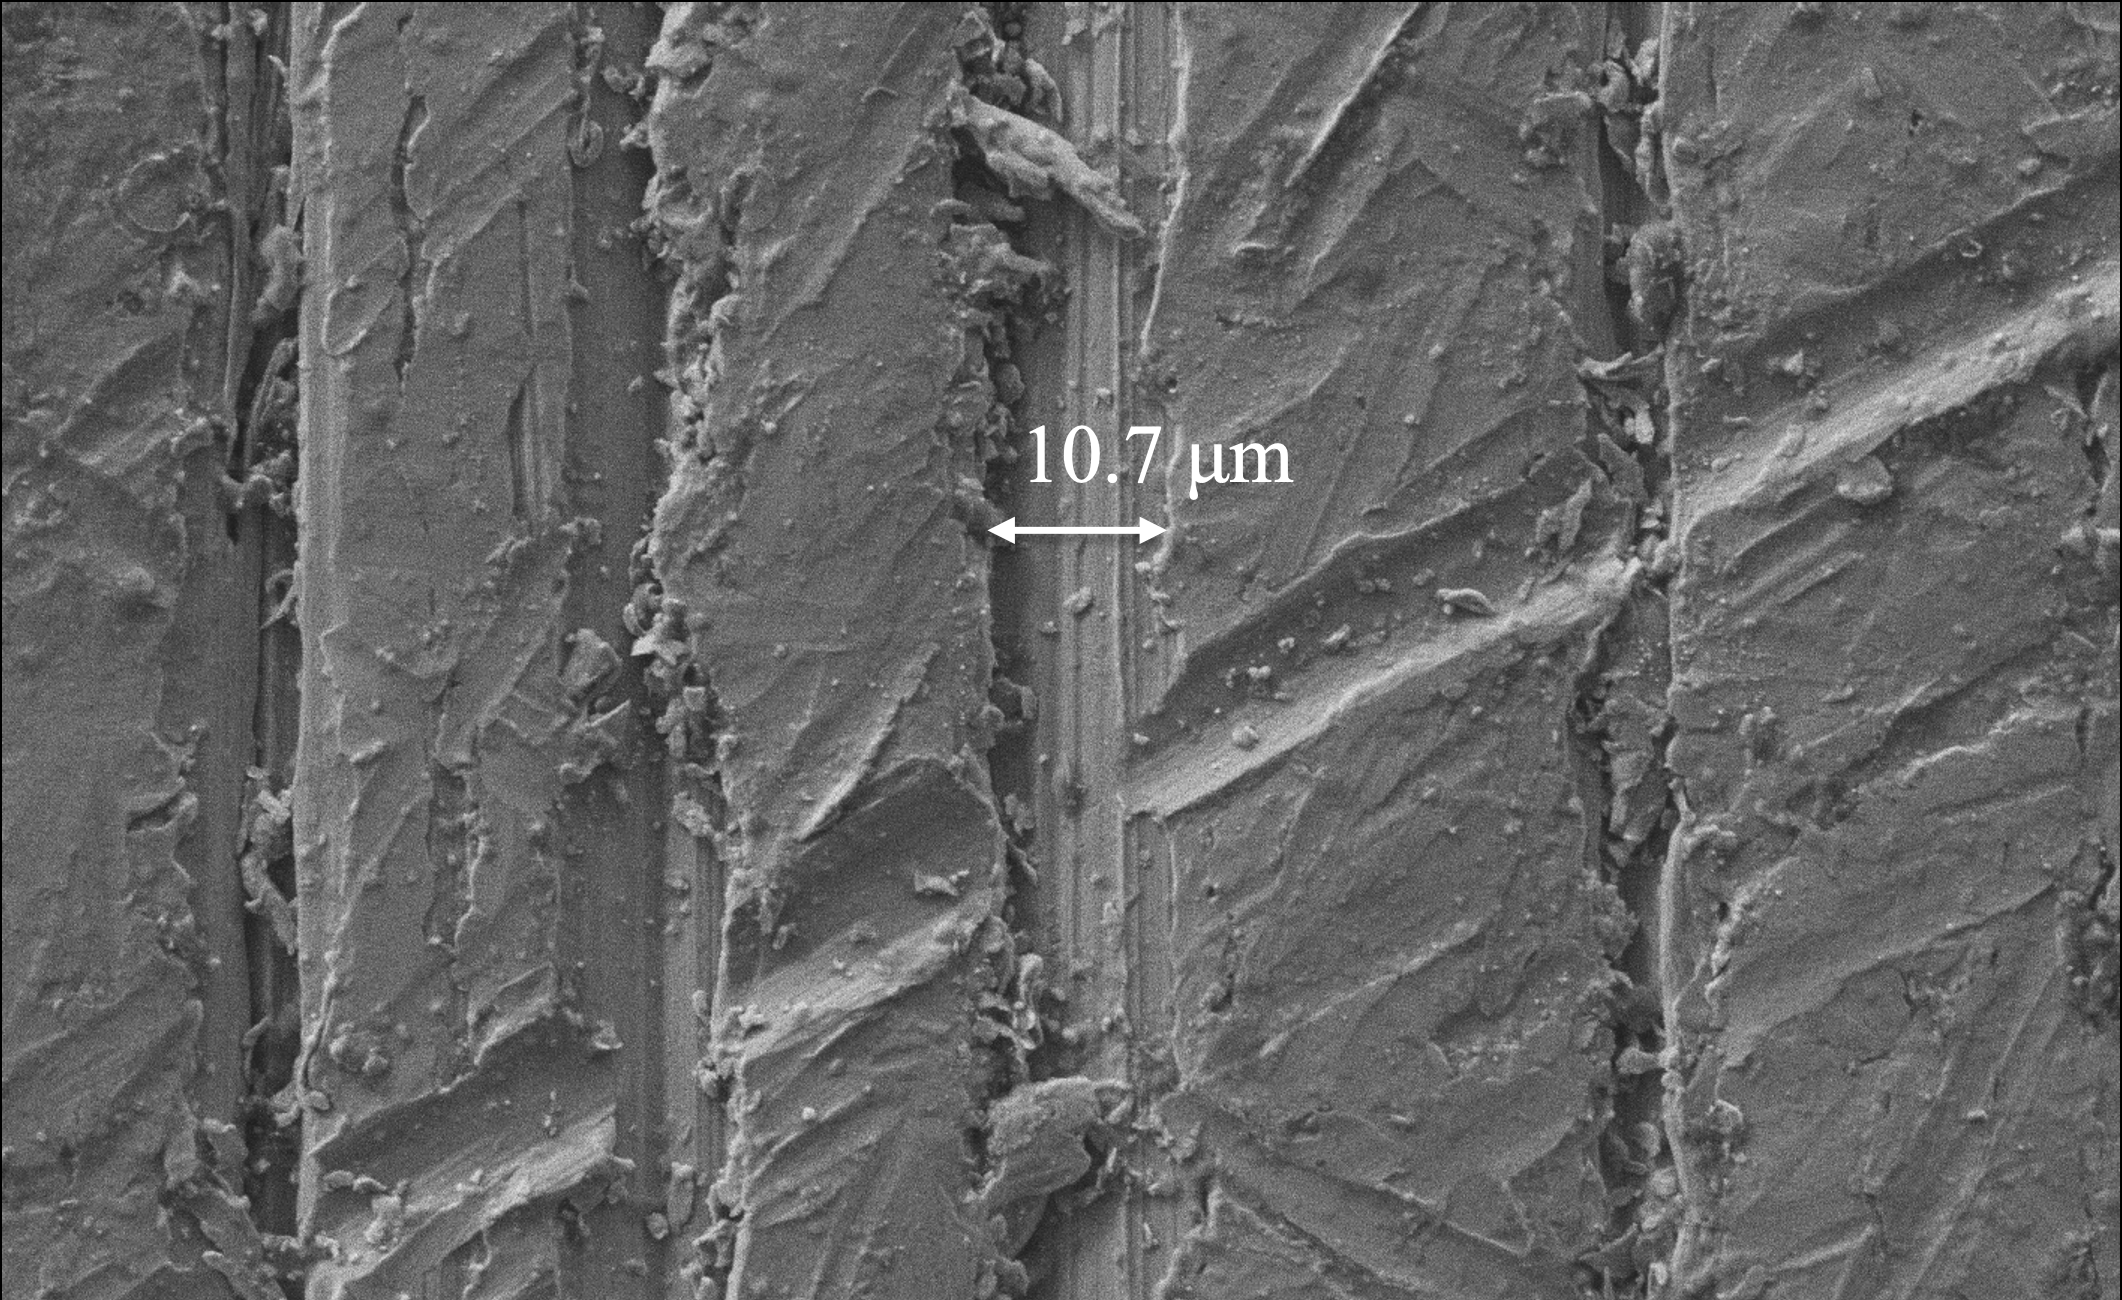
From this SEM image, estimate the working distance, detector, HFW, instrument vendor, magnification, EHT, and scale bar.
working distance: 7.211 mm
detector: SED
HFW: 127 µm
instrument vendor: other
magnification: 1 K X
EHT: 10 kV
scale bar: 30000 nm